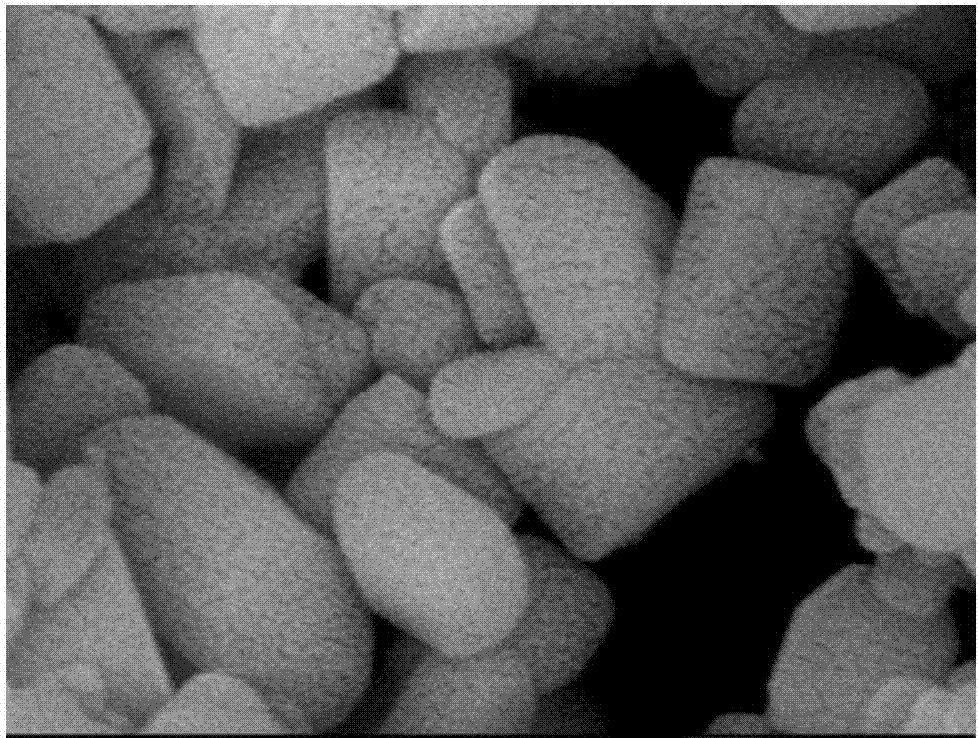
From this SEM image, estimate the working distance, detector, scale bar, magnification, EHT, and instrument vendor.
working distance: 8.1 mm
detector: SEI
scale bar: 100 nm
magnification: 90 K X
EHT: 8 kV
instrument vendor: JEOL